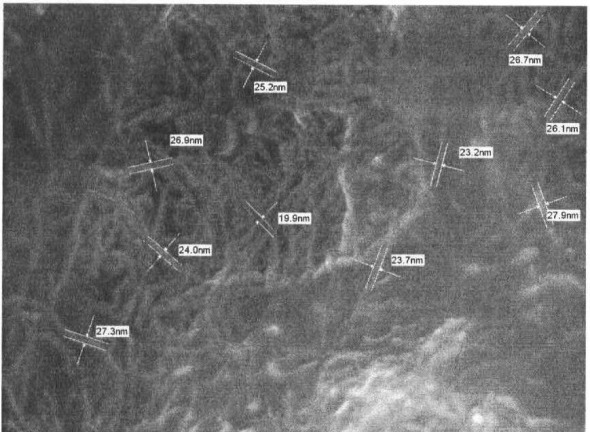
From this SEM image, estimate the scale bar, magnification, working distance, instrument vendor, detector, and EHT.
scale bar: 100 nm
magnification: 50 K X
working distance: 8 mm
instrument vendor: JEOL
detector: SEI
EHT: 10 kV